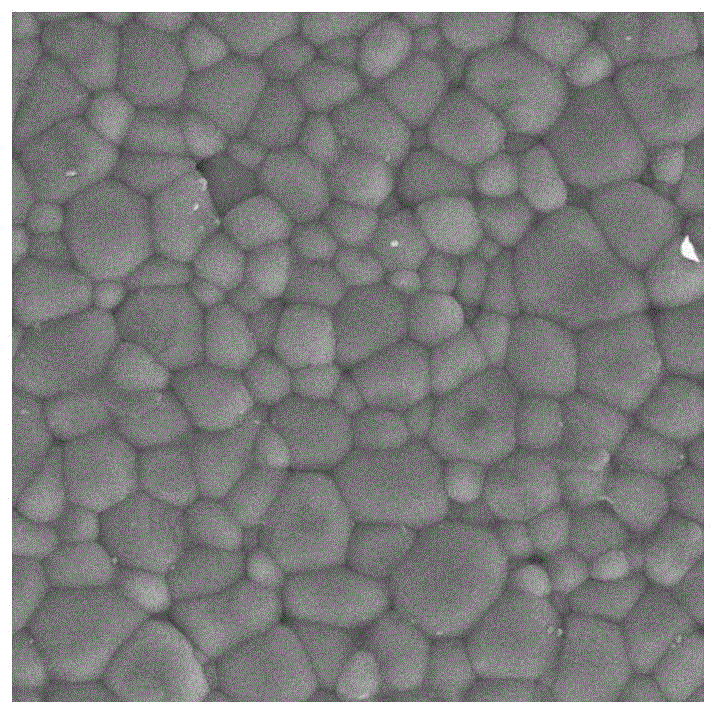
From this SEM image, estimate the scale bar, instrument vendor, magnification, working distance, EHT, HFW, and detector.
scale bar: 10000 nm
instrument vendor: Tescan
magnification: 5 K X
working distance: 9.16 mm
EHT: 30 kV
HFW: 56.9 µm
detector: SE